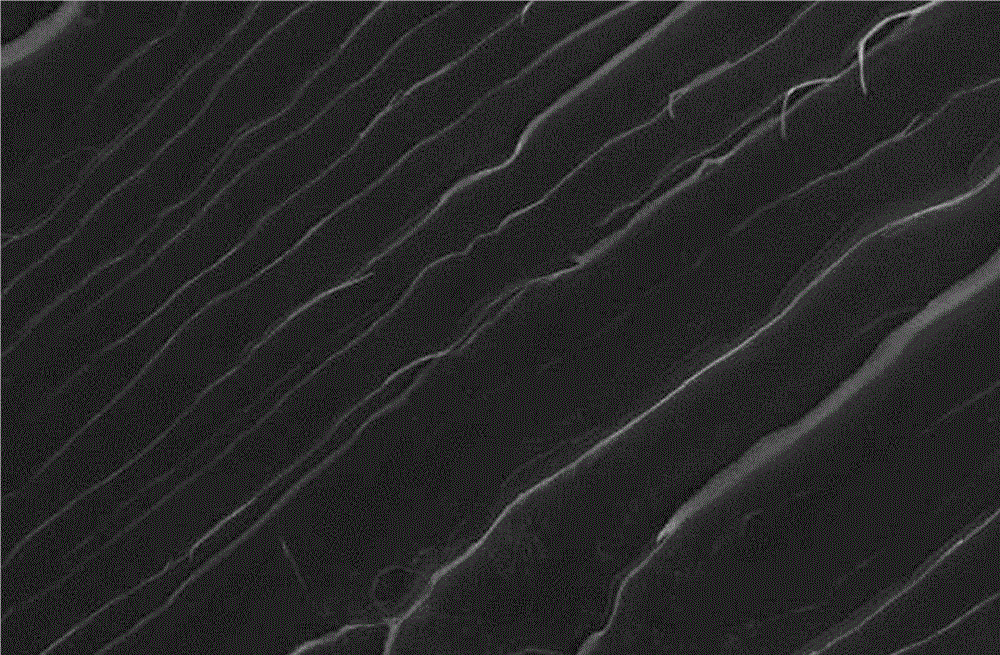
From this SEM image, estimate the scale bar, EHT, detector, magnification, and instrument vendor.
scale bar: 10000 nm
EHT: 20 kV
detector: SEI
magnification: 1 K X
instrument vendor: JEOL